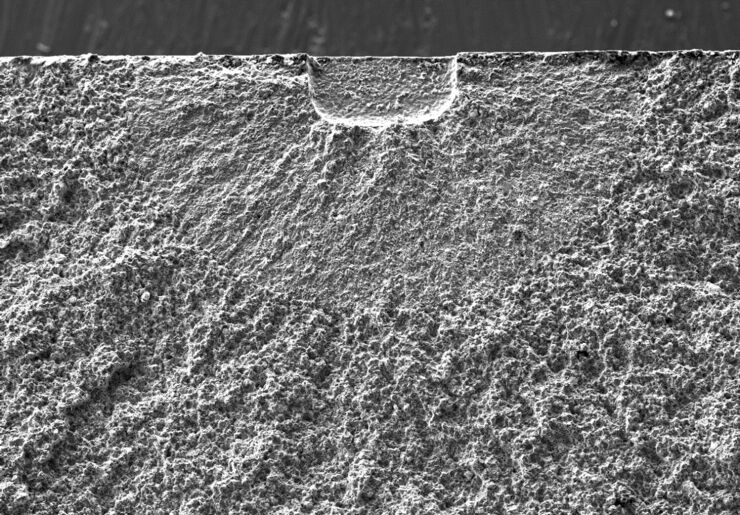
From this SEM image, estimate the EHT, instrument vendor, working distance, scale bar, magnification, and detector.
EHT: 20 kV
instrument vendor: Hitachi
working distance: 10 mm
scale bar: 500000 nm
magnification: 0.08 K X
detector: SE(M)